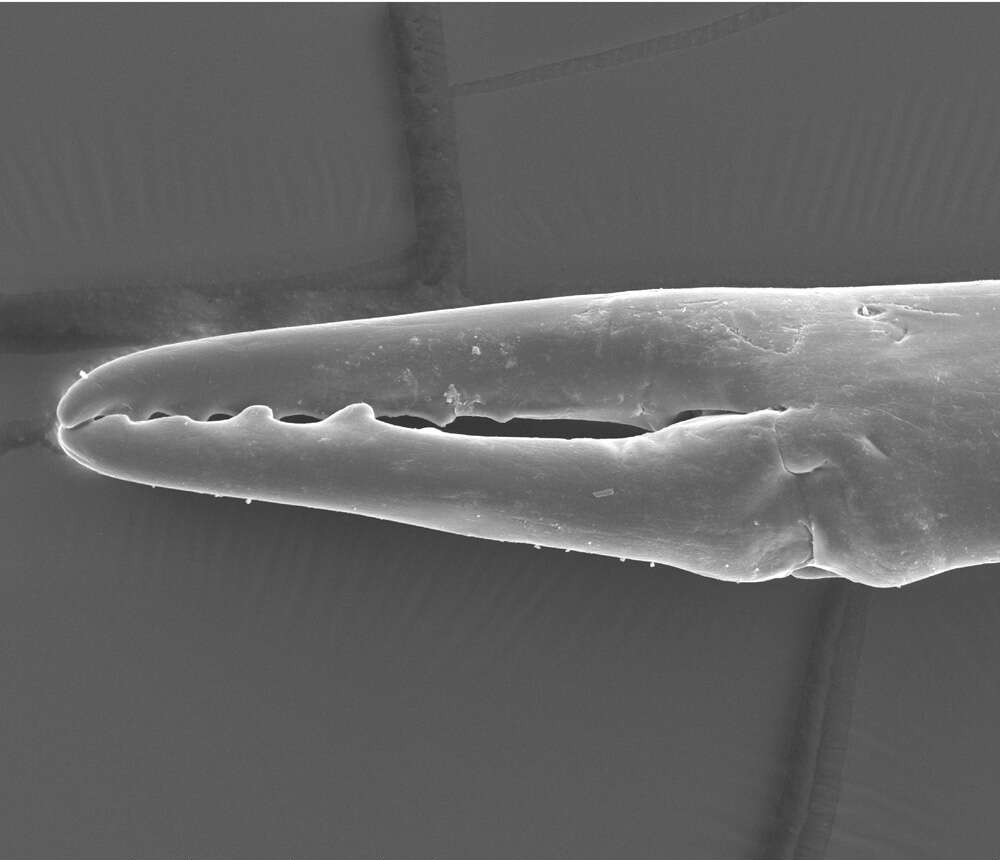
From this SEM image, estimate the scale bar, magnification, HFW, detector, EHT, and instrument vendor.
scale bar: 200000 nm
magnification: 0.341 K X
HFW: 370 µm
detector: ETD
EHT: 15 kV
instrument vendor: FEI